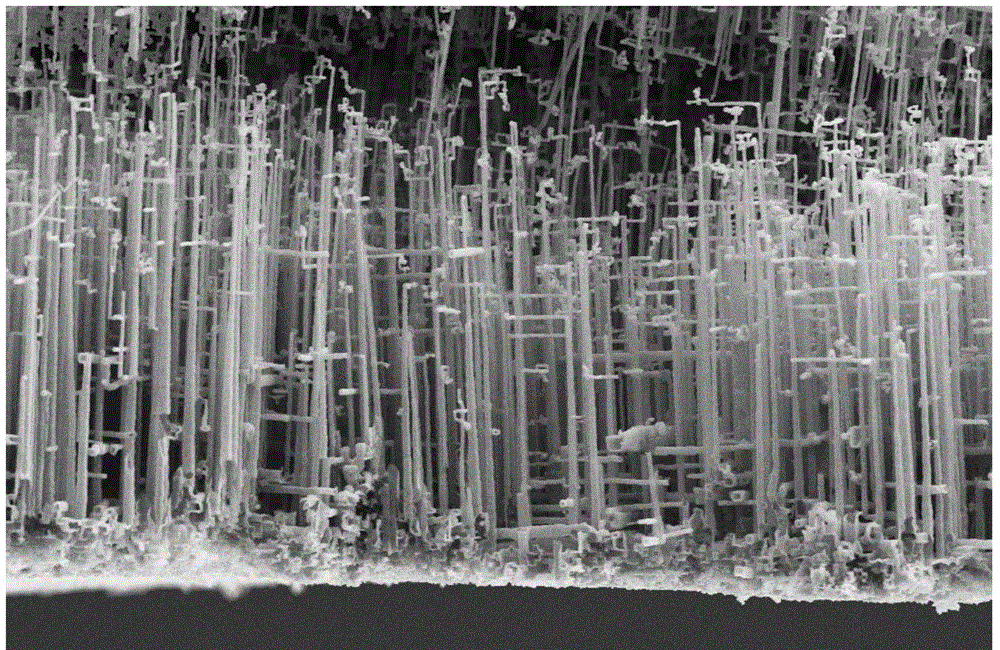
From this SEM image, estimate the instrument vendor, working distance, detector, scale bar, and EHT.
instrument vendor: Zeiss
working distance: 8 mm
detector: SE2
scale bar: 10000 nm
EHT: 10 kV